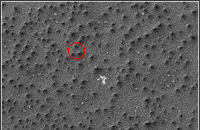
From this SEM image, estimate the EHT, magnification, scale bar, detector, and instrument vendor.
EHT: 10 kV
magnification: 0.4 K X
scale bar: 50000 nm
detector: SEI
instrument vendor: JEOL